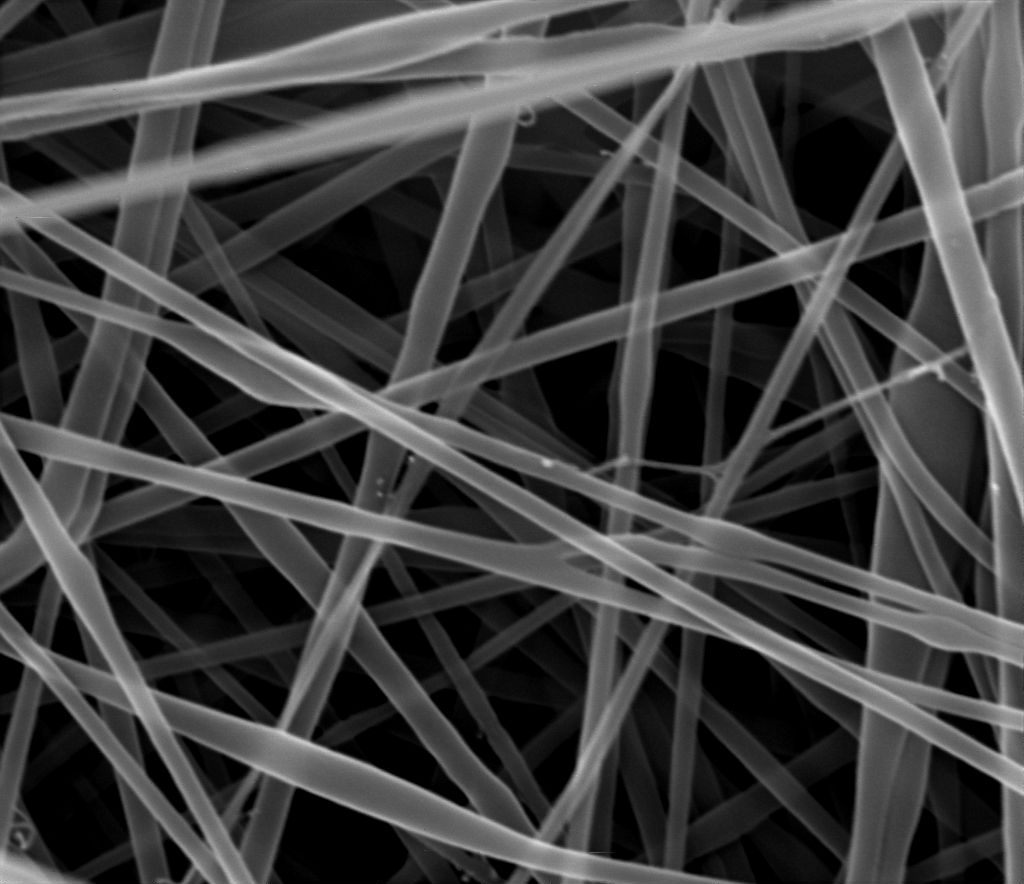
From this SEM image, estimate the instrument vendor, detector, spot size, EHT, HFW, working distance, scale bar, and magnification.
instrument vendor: FEI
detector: ETD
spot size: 3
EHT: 30 kV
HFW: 5.97 µm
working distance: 9.7 mm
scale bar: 1000 nm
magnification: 50 K X